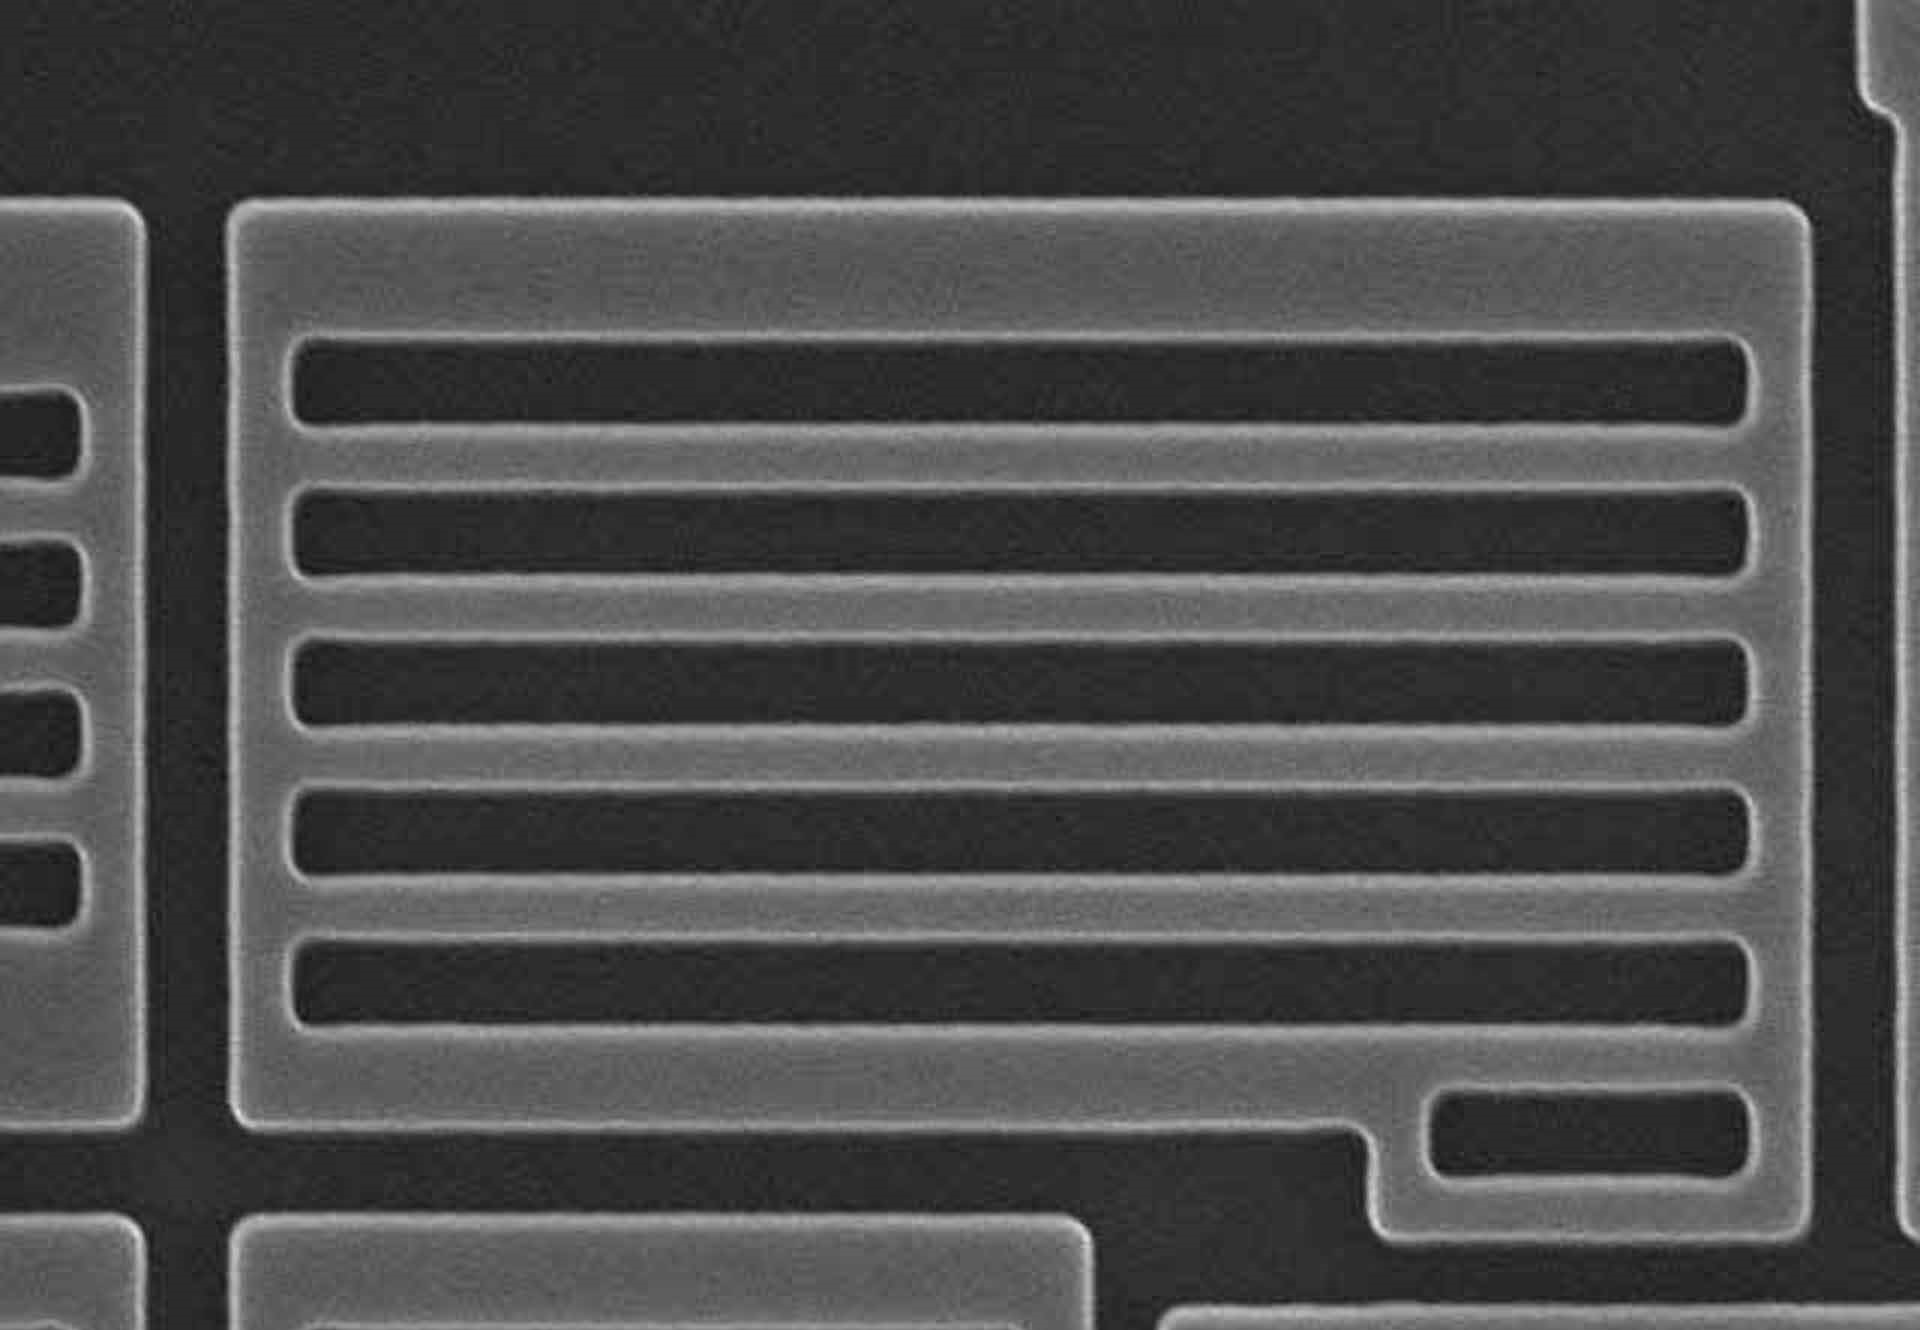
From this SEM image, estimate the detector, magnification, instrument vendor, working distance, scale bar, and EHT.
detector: SE(M)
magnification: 25 K X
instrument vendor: Hitachi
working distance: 18.8 mm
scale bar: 2000 nm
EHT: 15 kV